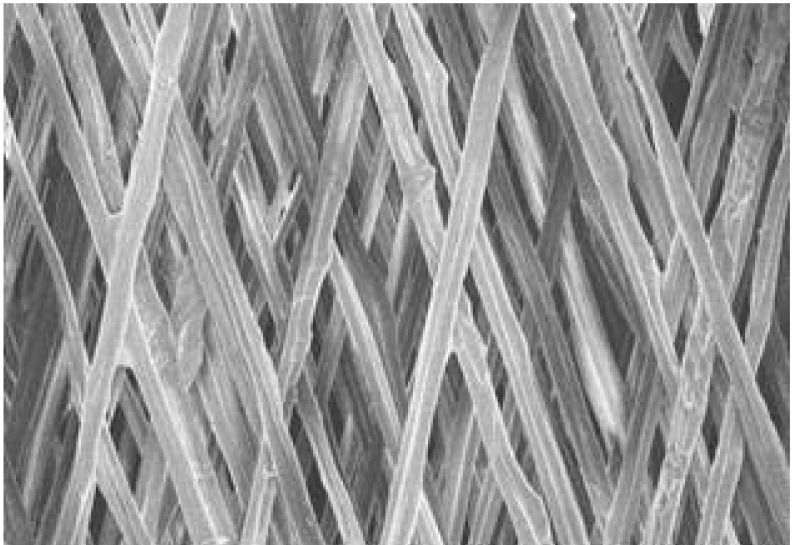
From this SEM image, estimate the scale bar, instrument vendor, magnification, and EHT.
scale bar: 500000 nm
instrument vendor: Hitachi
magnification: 0.1 K X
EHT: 15 kV